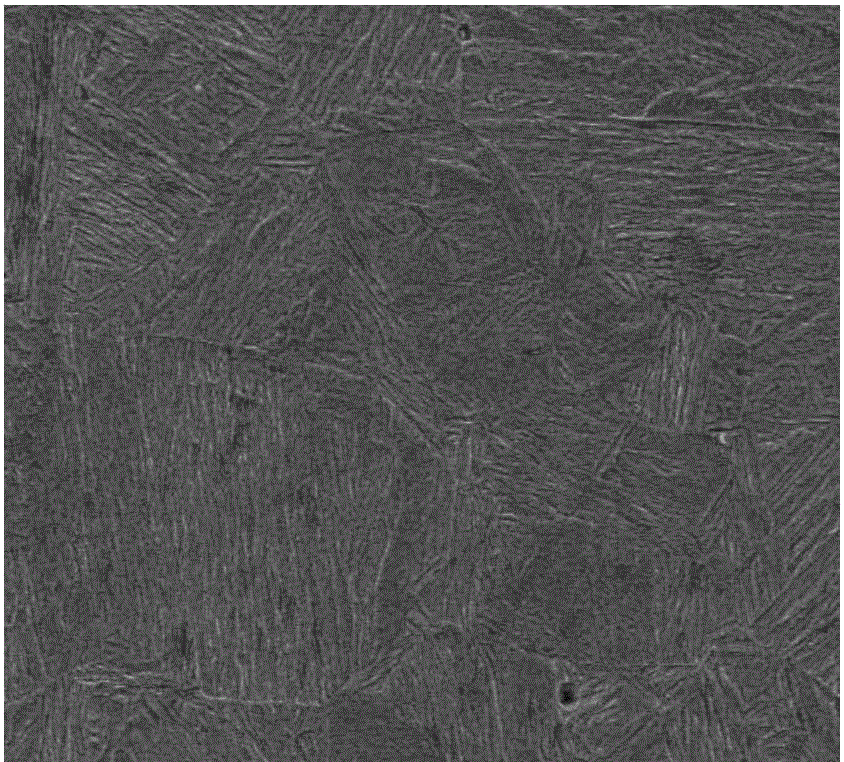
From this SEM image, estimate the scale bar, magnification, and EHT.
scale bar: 40000 nm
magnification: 0.5 K X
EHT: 20 kV